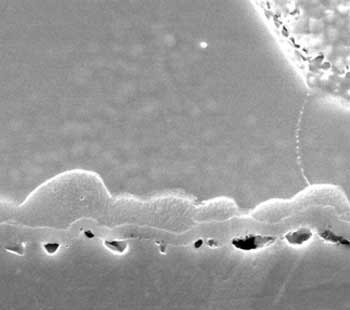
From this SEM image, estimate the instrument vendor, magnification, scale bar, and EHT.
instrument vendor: JEOL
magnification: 10 K X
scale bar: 3000 nm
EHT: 15 kV